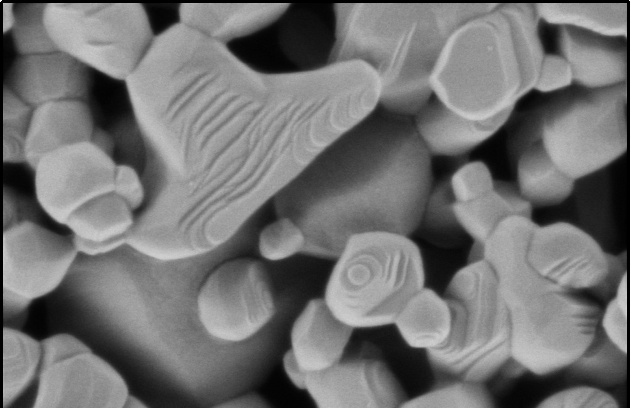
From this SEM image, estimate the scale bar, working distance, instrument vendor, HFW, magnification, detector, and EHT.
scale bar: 500 nm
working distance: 3.1 mm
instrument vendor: Thermo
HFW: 1.27 µm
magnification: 100 K X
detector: T2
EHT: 0.5 kV